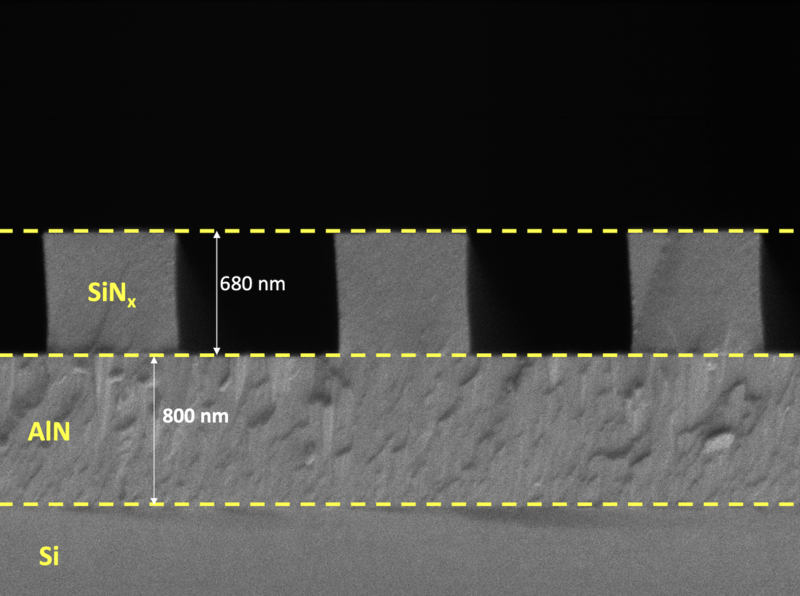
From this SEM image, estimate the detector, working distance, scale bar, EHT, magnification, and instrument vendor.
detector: SEI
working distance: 7.2 mm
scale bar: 1000 nm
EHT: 1 kV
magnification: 27 K X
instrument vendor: JEOL